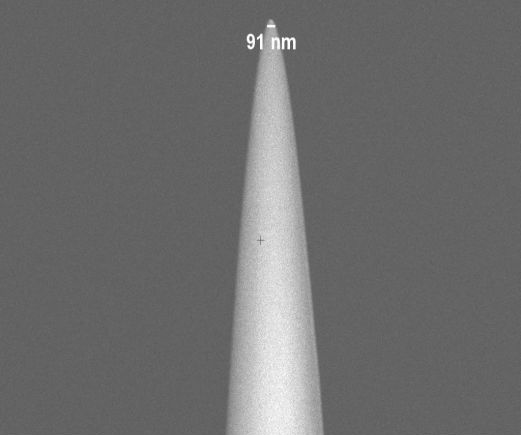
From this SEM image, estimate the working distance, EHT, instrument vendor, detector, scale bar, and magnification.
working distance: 4.2 mm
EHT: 2 kV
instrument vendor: Zeiss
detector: InLens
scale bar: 2000 nm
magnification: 20 K X